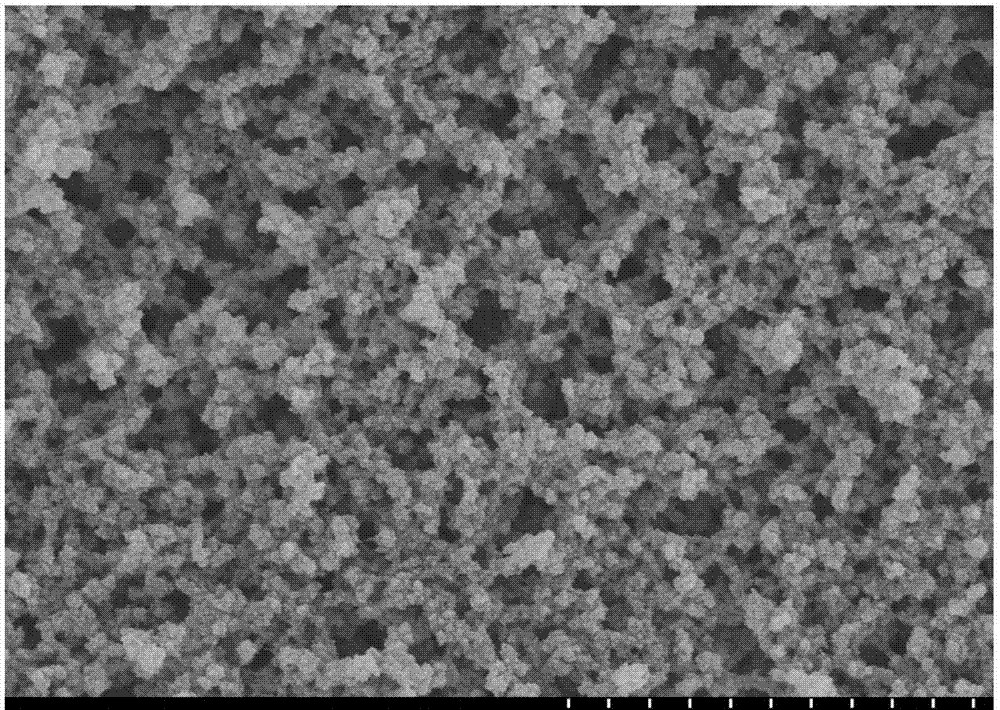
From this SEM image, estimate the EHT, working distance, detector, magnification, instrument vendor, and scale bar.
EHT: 5 kV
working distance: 9 mm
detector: SE(M)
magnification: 13 K X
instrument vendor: Hitachi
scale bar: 4000 nm